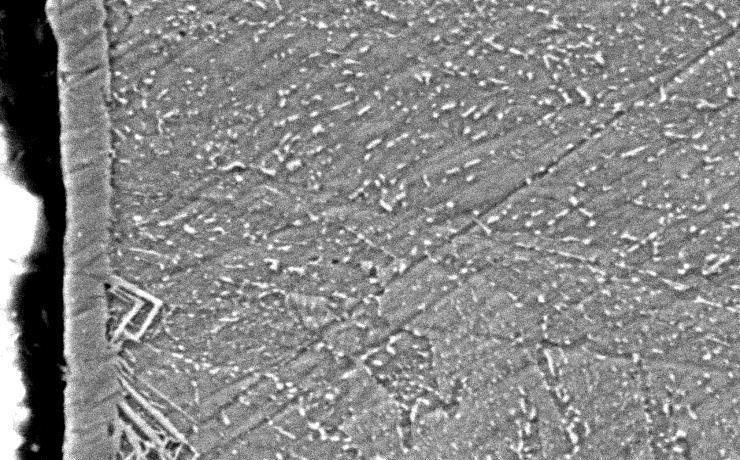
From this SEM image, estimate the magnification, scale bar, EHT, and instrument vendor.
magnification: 5 K X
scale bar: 2000 nm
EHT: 10 kV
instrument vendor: JEOL